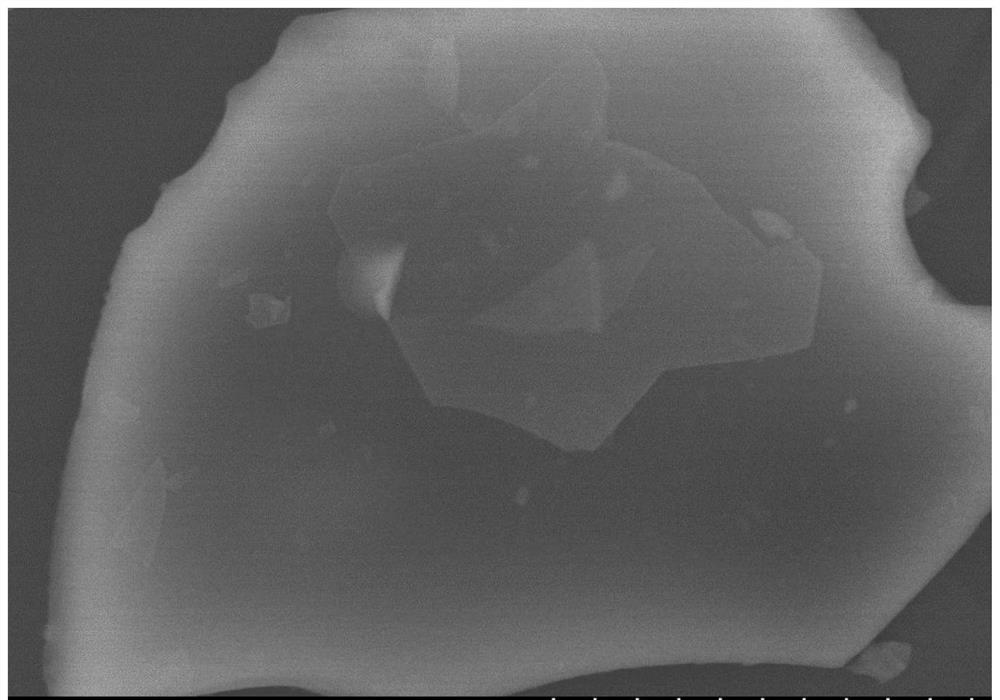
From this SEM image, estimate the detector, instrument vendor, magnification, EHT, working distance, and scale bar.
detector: SE(M)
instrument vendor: Hitachi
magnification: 18 K X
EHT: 10 kV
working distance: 9 mm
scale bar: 3000 nm